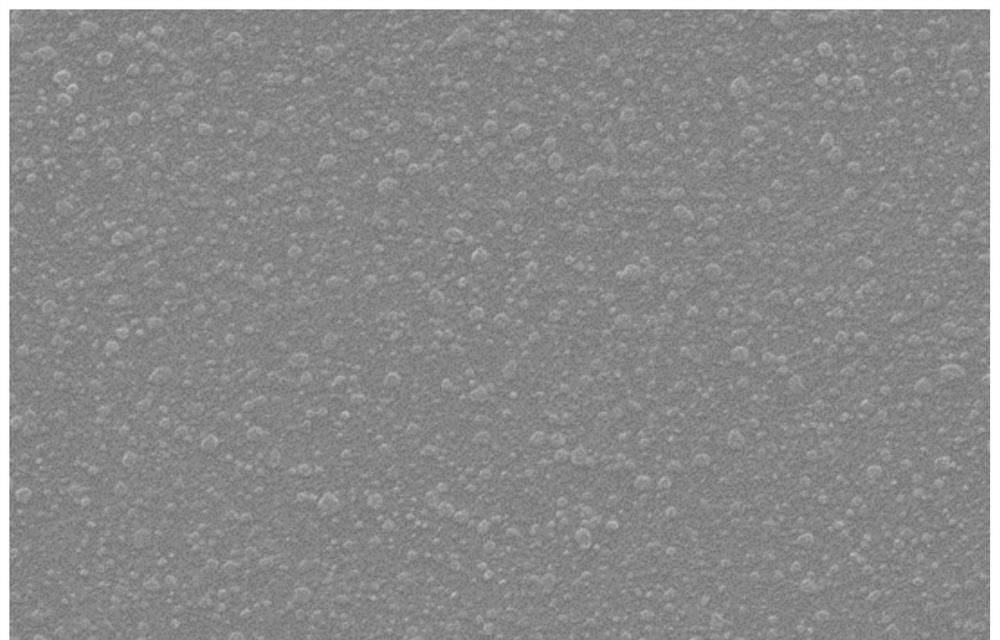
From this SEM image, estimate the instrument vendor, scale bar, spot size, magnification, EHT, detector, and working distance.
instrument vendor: FEI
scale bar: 20000 nm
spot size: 3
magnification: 1 K X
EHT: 15 kV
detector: SE2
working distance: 9.6 mm